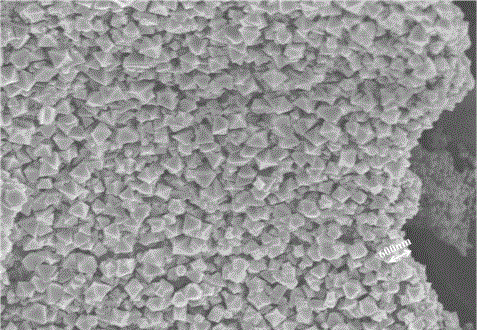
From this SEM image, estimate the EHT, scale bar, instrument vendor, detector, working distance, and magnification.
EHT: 5 kV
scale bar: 5000 nm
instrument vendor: Hitachi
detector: SE(M)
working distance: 10.6 mm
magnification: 8 K X